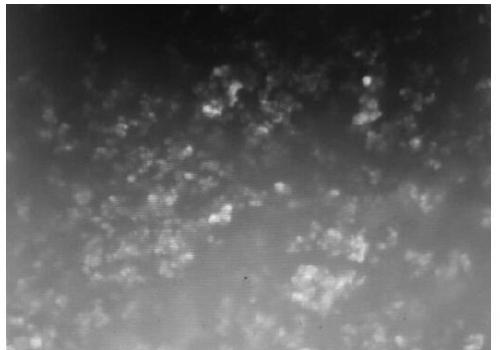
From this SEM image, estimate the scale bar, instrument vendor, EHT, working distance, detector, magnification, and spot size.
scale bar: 3000 nm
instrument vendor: FEI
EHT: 5 kV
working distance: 9.7 mm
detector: GSED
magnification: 30 K X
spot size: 3.9